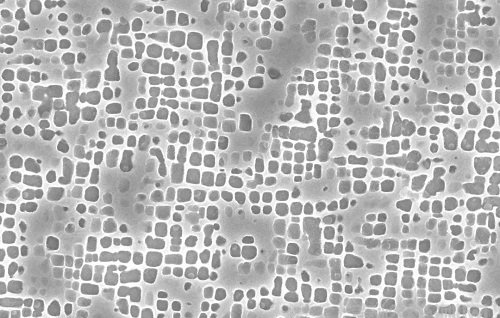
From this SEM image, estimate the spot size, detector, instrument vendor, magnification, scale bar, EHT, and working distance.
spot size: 3.5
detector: SE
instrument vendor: FEI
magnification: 20 K X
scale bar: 3000 nm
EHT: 20 kV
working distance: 10.5 mm